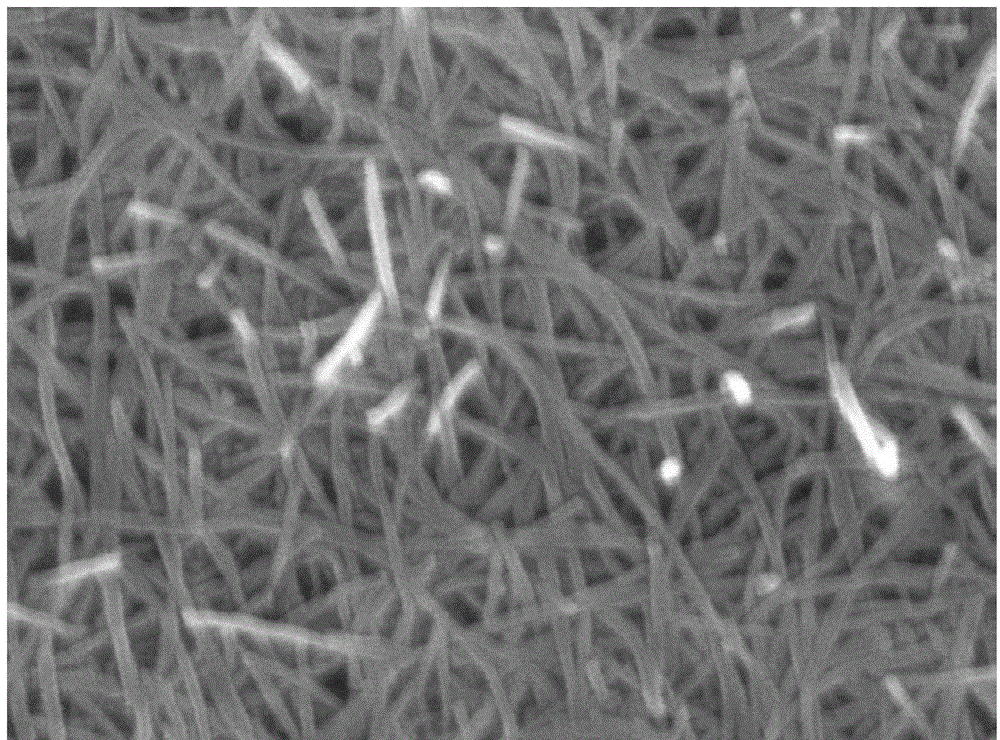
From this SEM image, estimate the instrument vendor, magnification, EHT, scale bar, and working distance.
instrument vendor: Hitachi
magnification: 80 K X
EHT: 10 kV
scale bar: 500 nm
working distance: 8.7 mm